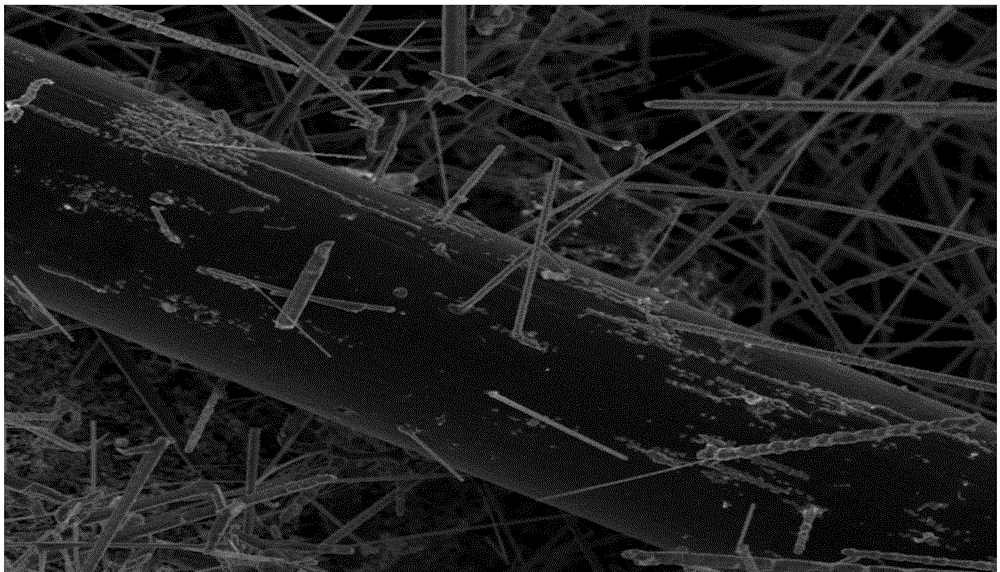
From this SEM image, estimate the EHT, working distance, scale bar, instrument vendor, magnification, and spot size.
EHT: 3 kV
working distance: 6.3 mm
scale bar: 5000 nm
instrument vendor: FEI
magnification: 15 K X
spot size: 3